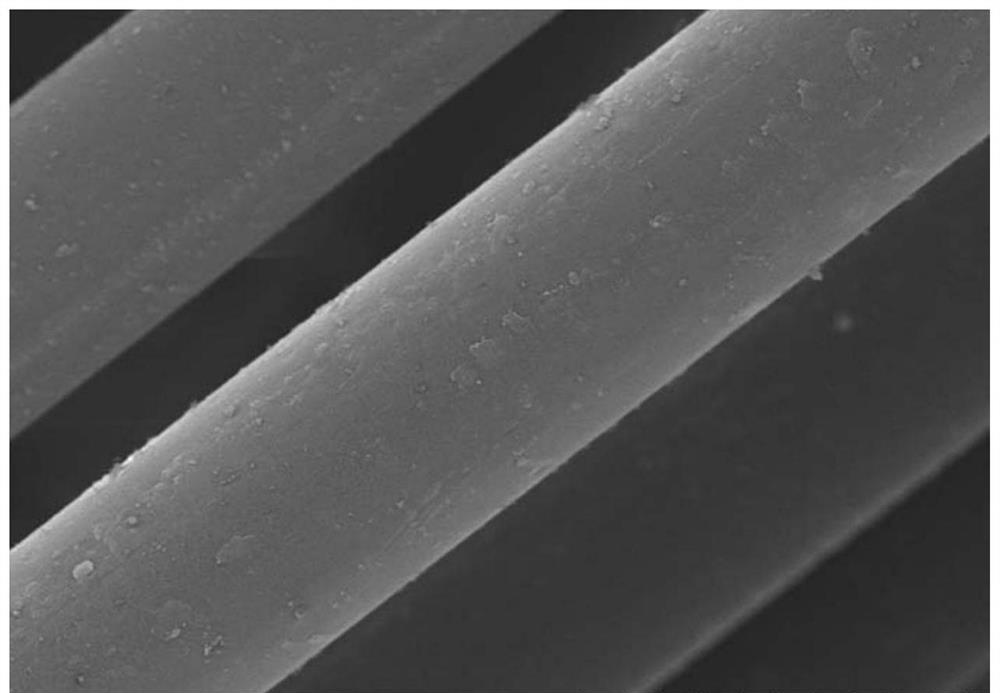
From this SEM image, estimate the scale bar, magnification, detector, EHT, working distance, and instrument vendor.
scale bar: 10000 nm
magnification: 5 K X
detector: SE(M)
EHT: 10 kV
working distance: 7.7 mm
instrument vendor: Hitachi